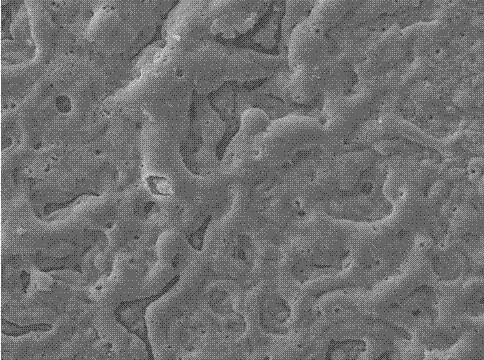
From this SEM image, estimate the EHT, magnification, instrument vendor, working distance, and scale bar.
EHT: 15 kV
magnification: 1 K X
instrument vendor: Tescan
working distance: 14.3 mm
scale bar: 20000 nm